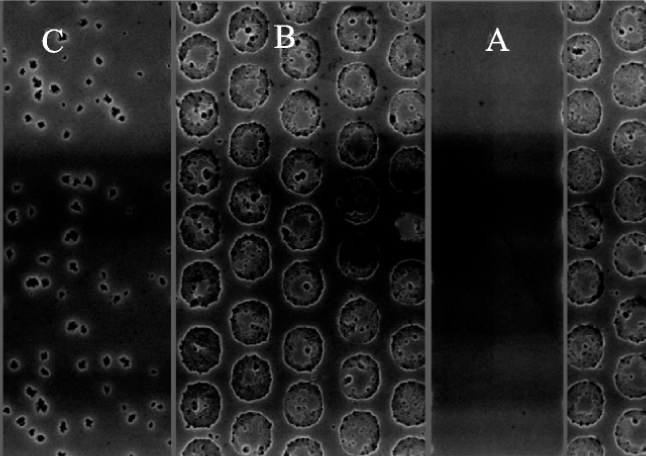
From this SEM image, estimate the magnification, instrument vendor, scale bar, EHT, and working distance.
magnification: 6.5 K X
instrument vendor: FEI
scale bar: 5000 nm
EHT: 10 kV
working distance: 5.1 mm